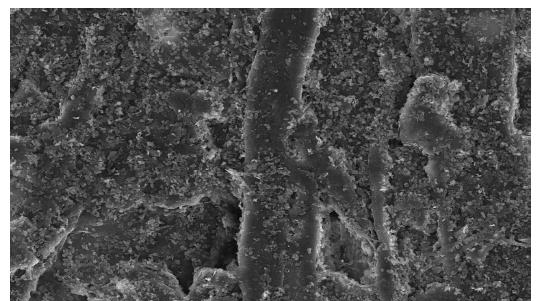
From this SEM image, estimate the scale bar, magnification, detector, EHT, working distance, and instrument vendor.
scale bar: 50000 nm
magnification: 1 K X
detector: SE(U)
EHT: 15 kV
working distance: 12.2 mm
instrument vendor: Hitachi